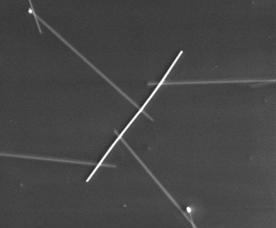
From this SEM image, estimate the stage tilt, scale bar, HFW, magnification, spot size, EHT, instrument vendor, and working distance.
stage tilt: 0°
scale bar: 5000 nm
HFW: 46.8 µm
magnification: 6.5 K X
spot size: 2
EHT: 10 kV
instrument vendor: FEI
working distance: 5.03 mm